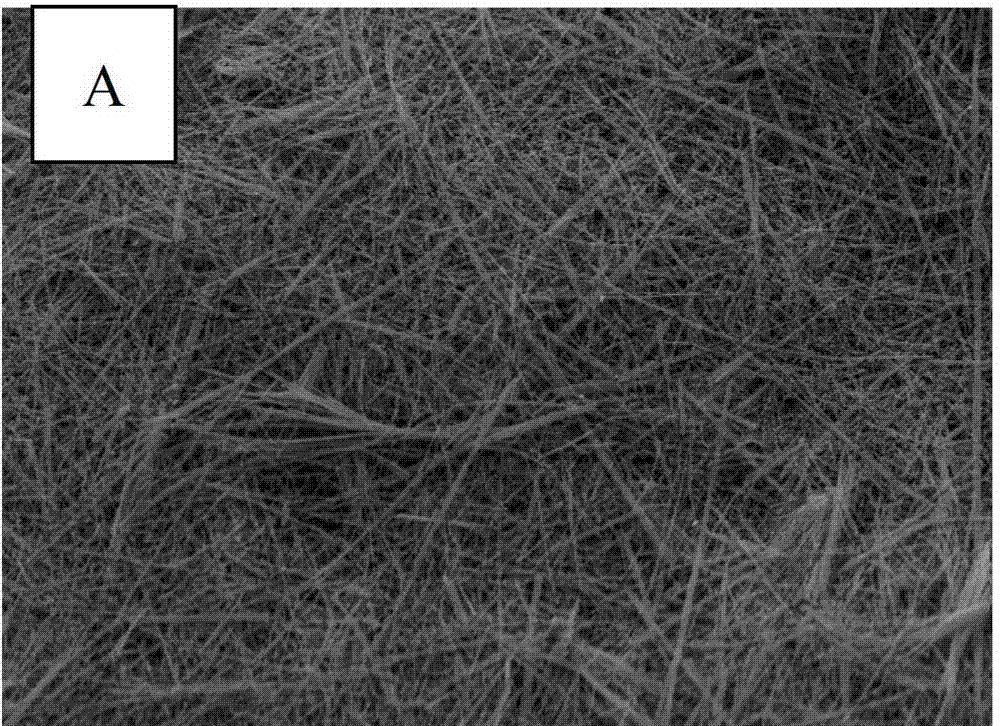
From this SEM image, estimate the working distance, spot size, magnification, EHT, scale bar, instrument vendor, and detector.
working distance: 8.1 mm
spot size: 2.5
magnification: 5 K X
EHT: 12 kV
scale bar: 10000 nm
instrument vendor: FEI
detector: SE